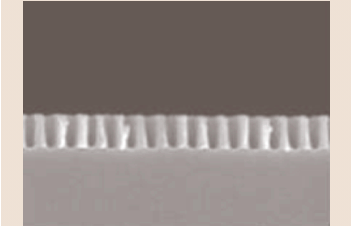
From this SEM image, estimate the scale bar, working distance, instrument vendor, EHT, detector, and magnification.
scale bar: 100 nm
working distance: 6.7 mm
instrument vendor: JEOL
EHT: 3 kV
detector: SEI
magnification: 70 K X